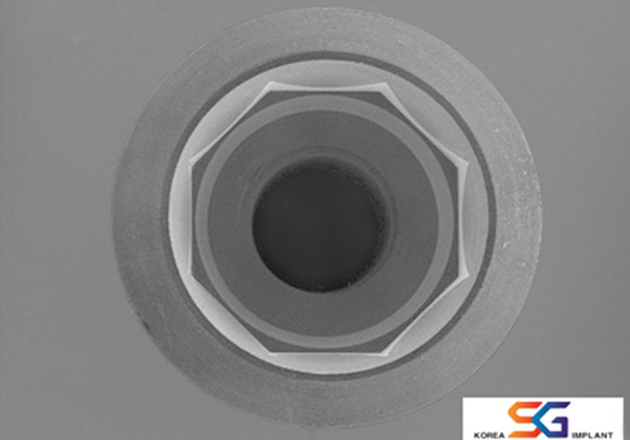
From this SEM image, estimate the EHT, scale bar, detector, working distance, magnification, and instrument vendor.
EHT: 15 kV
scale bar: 3000000 nm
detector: SE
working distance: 30 mm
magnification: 0.017 K X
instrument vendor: Hitachi